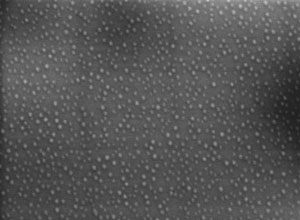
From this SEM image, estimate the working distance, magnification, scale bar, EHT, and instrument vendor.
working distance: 7.8 mm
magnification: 100 K X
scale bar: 500 nm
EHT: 10 kV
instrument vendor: Hitachi